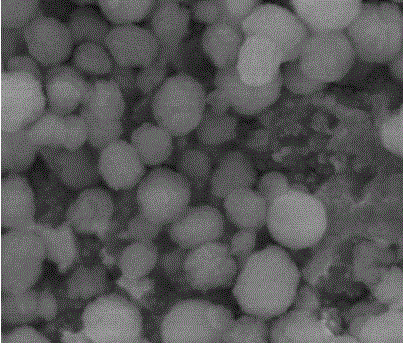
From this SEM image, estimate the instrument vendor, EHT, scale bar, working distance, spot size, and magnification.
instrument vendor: FEI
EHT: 20 kV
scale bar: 1000 nm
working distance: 6.6 mm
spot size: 2.5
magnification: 50 K X